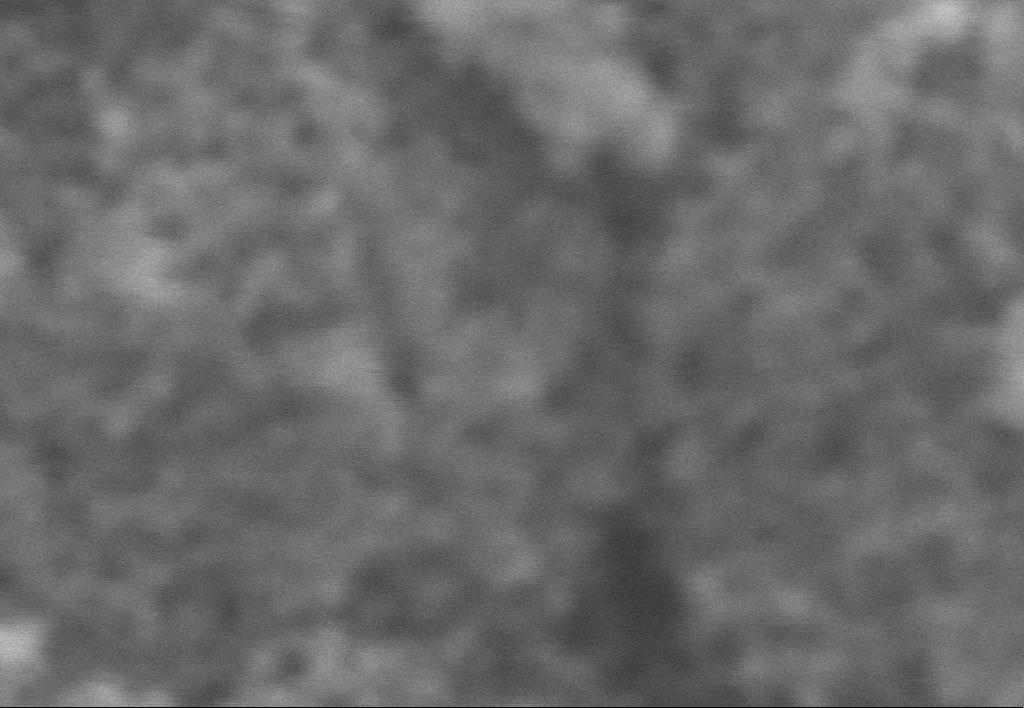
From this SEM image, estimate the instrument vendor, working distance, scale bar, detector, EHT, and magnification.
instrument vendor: Zeiss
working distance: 12 mm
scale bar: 100 nm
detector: SE1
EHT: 25 kV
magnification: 300 K X